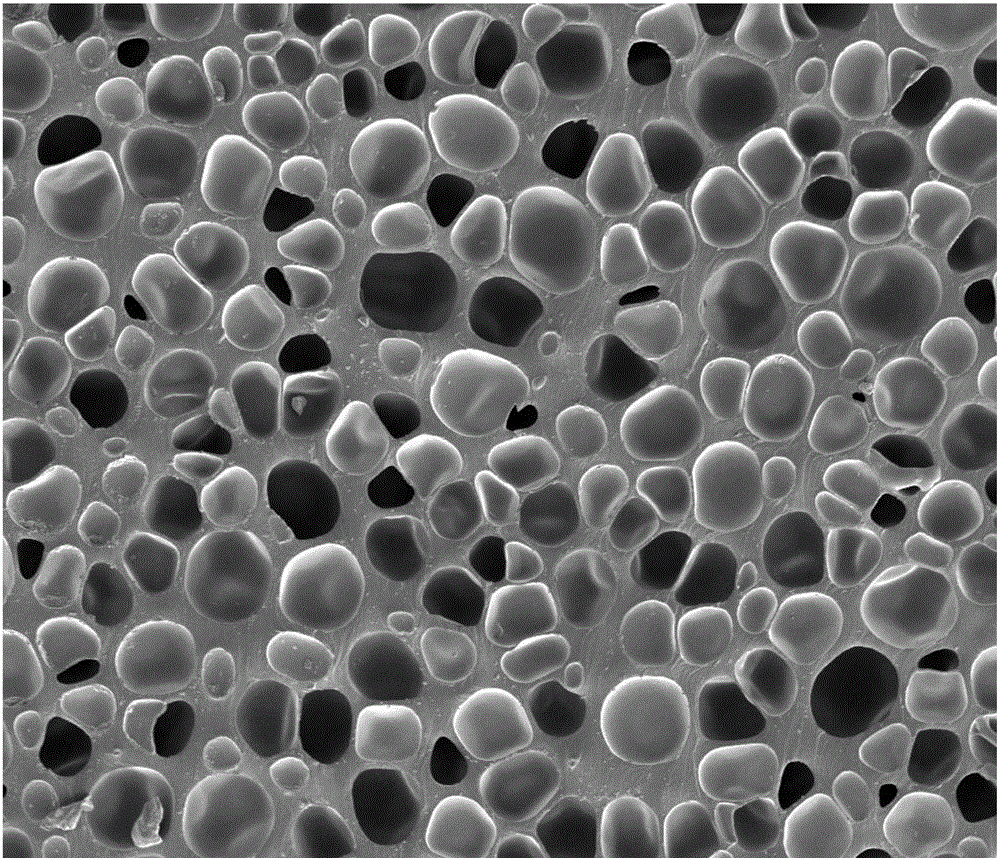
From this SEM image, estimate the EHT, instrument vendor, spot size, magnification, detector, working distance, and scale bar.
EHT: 3 kV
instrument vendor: FEI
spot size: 3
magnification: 0.3 K X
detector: ETD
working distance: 5 mm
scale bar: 200000 nm